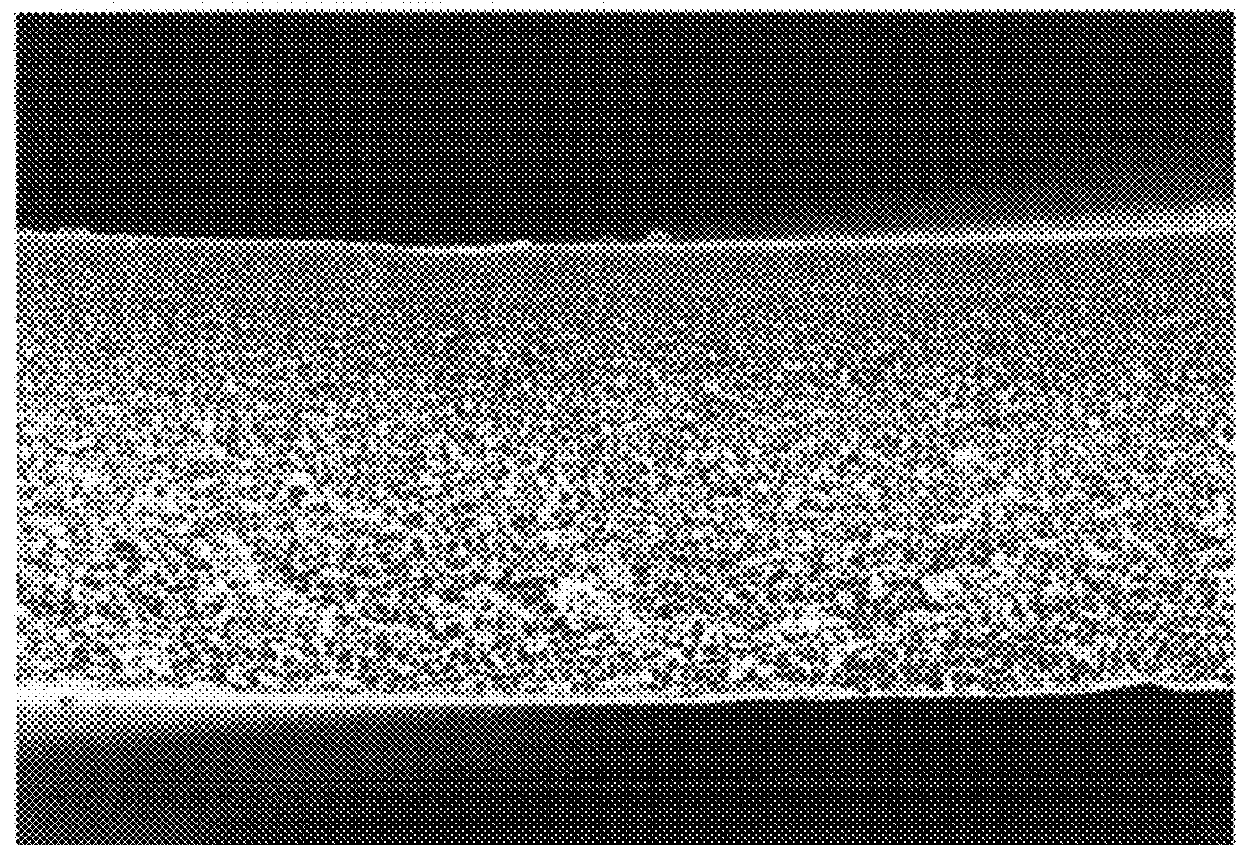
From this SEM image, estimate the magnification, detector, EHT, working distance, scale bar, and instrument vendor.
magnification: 2 K X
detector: SE(M)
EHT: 15 kV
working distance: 8.2 mm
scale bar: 20000 nm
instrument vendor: Hitachi